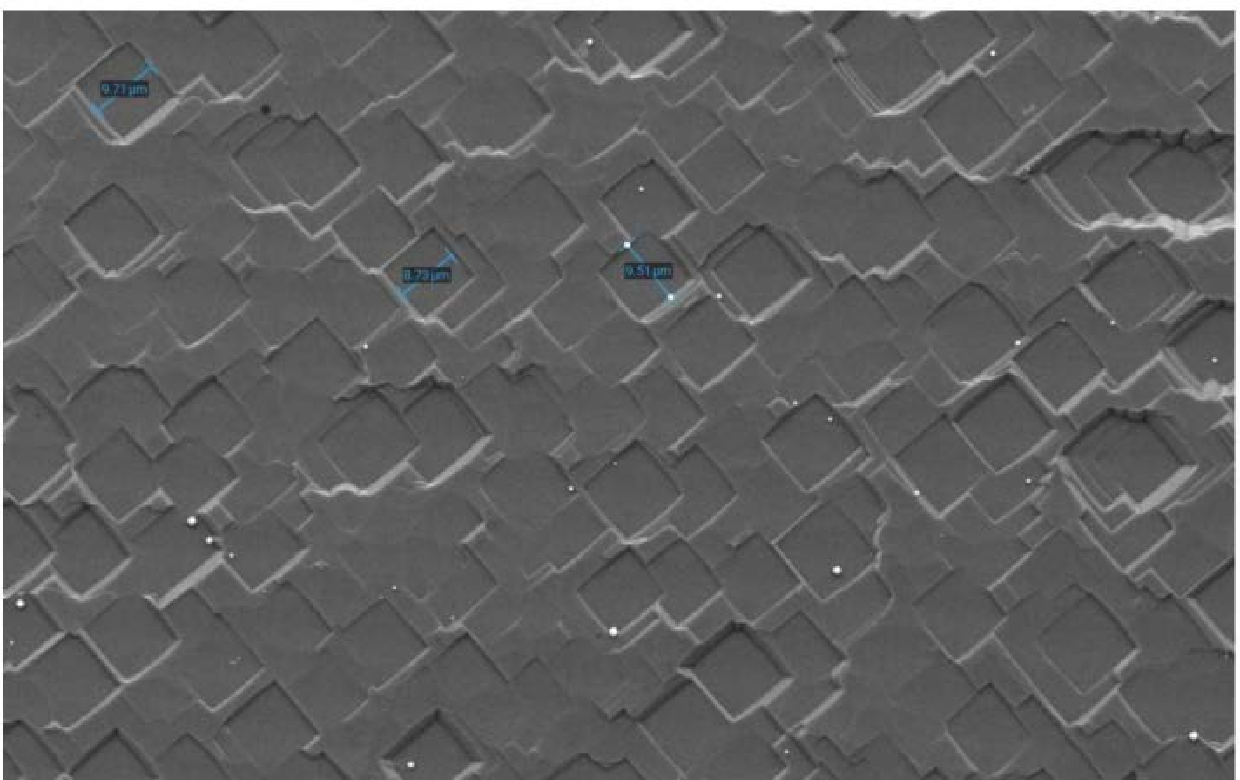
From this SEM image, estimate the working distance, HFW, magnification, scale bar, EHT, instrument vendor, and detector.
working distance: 7.252 mm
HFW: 173 µm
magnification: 3 K X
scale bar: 50000 nm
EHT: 10 kV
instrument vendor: other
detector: SED